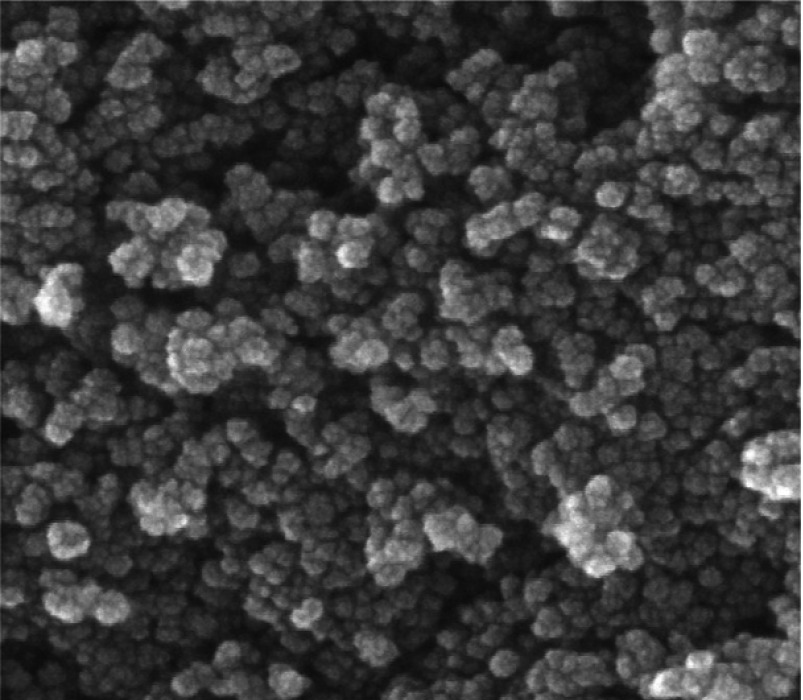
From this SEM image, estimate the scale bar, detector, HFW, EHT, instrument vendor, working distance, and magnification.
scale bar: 100 nm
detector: InBeam SE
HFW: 0.593 µm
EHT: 15 kV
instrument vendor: Tescan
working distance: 5.18 mm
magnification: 350 K X